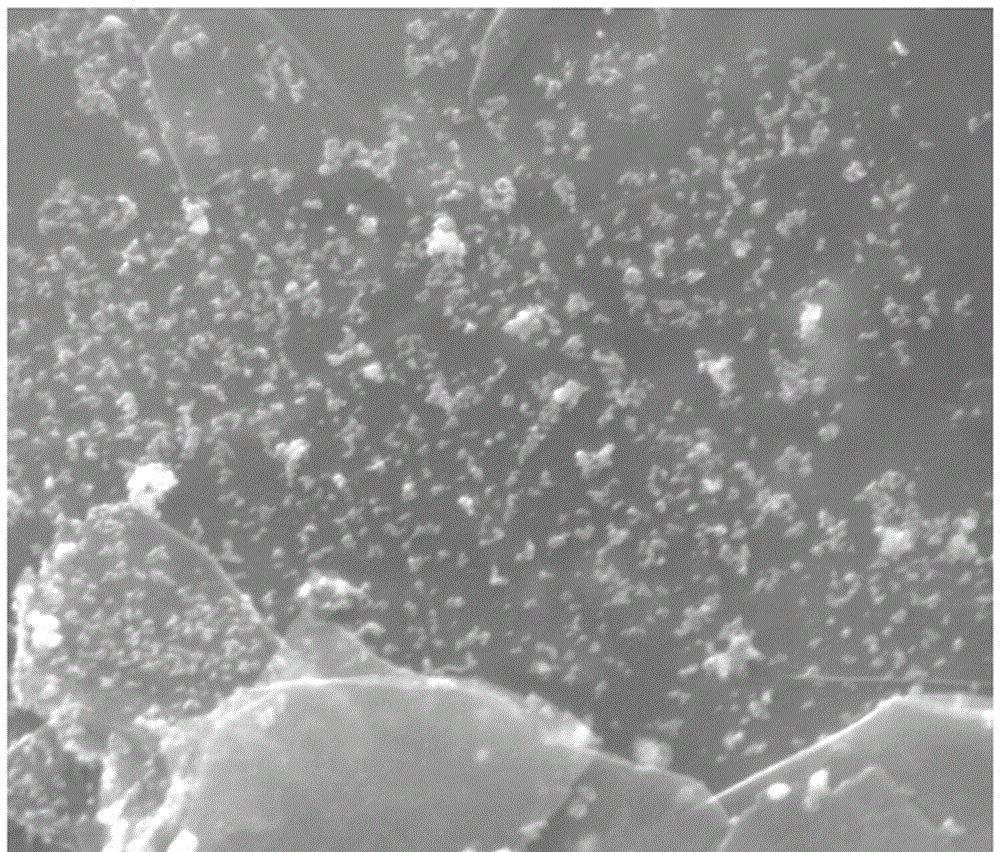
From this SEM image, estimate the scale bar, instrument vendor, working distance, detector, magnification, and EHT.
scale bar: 1000 nm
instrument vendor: FEI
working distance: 9.3 mm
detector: ETD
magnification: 100 K X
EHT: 20 kV